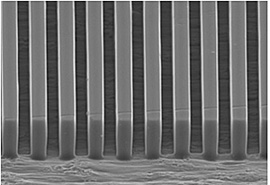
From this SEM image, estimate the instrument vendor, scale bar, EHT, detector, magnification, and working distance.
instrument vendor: Hitachi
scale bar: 30000 nm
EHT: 16 kV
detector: SE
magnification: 1.5 K X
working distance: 33.3 mm